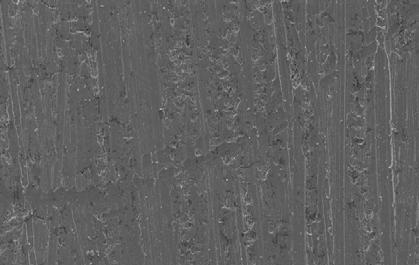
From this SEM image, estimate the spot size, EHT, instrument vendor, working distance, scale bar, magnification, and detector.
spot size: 4.4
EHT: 20 kV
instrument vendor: FEI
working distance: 10.4 mm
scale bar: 200000 nm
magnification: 0.2 K X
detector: SE